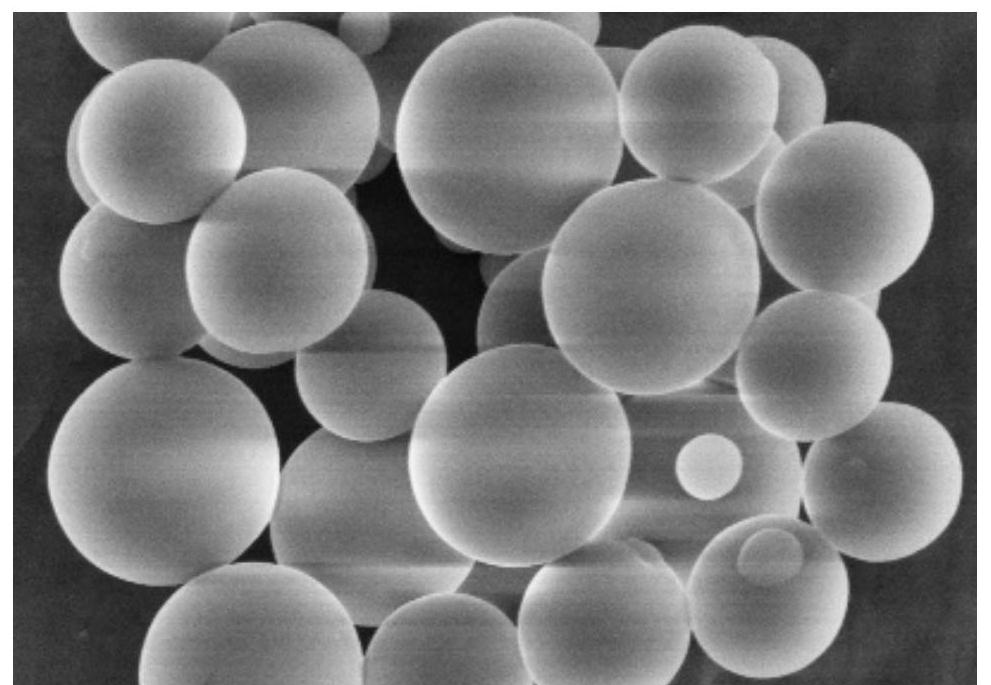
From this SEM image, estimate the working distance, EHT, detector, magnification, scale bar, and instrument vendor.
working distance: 9.3 mm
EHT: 5 kV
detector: SE(M,LA0)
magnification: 25 K X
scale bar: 2000 nm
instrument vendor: Hitachi